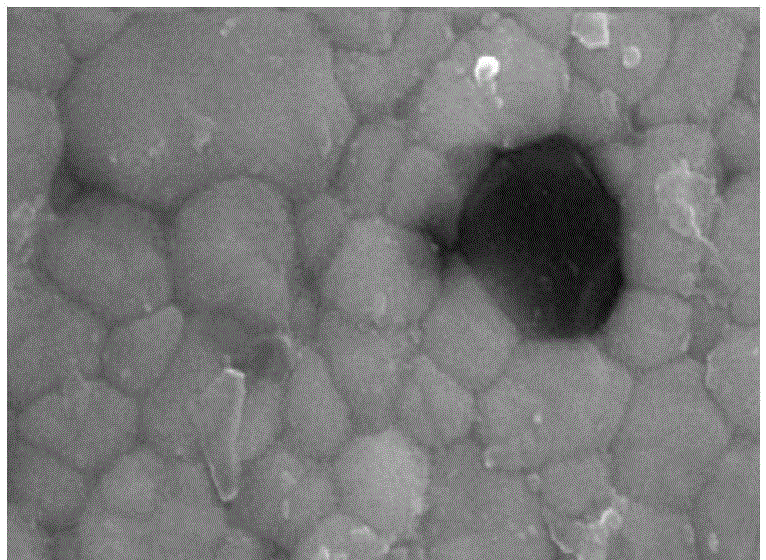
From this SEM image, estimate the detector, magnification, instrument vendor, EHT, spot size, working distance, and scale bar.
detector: SE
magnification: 40 K X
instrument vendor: FEI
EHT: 20 kV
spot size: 4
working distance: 6.3 mm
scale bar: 500 nm